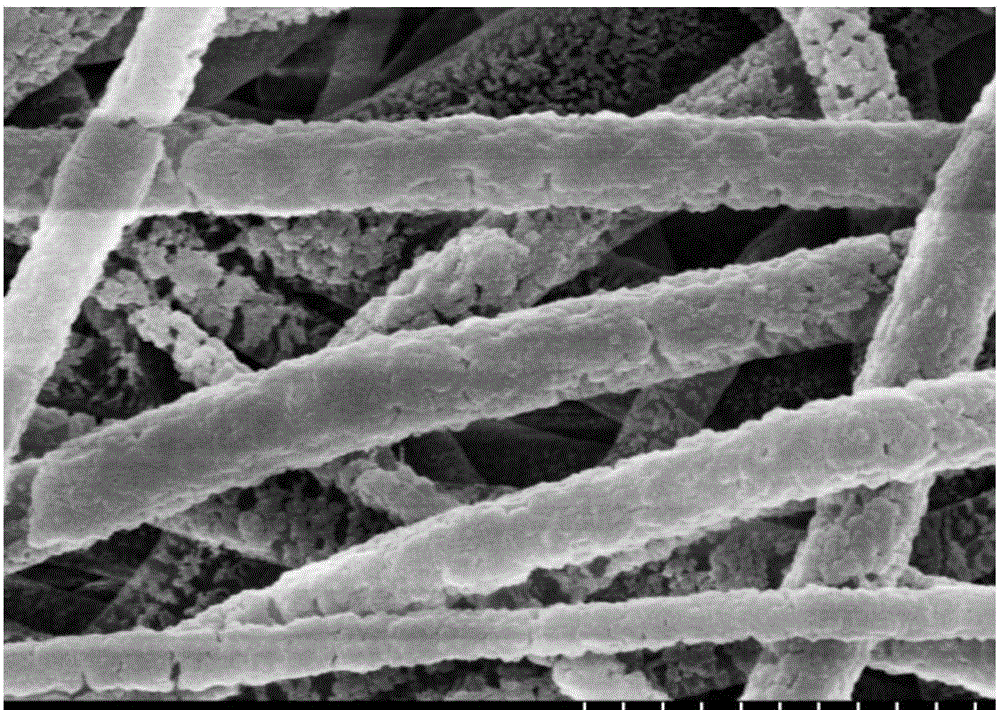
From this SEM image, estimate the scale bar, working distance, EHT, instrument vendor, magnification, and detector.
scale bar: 2000 nm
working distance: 9.6 mm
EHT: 5 kV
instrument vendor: Hitachi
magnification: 25 K X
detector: SE(U)